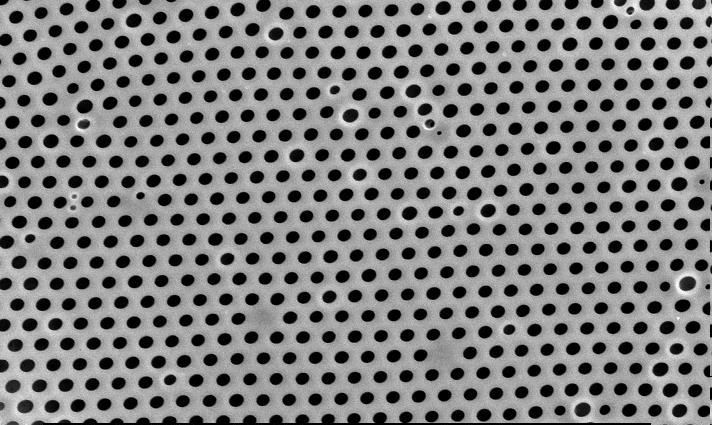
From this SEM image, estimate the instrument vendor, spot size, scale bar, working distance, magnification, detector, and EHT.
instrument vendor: FEI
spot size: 4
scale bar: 10000 nm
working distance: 6 mm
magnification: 5 K X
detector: SE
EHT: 25 kV